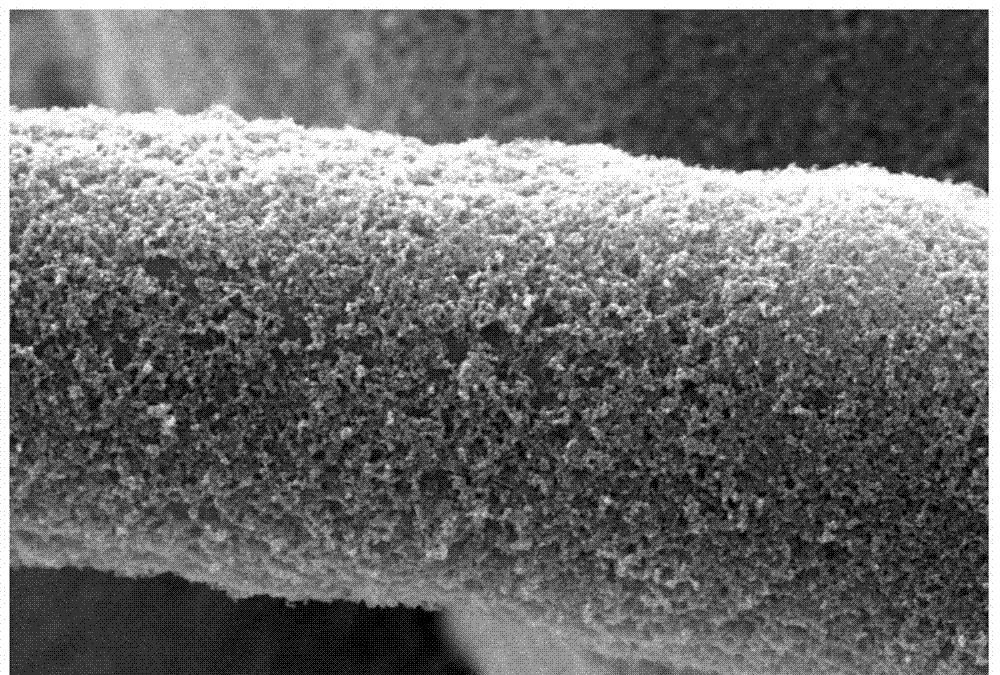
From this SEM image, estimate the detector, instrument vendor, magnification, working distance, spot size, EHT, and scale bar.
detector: SEI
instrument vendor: JEOL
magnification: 5 K X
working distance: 8 mm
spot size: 40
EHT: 20 kV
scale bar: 5000 nm